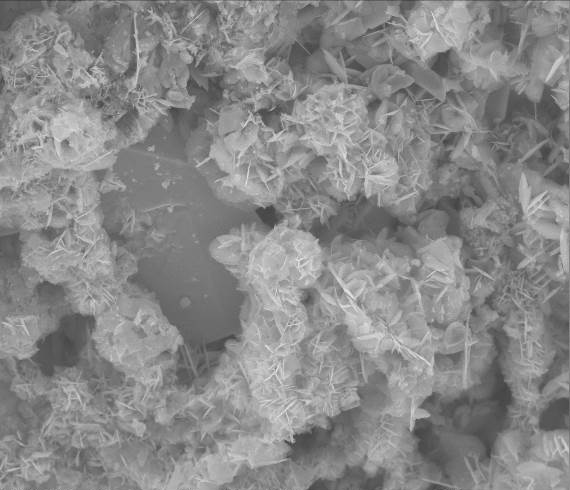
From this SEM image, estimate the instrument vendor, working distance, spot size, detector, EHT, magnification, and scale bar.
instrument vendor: FEI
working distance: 14.6 mm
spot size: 3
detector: ETD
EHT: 30 kV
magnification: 1.313 K X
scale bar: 50000 nm